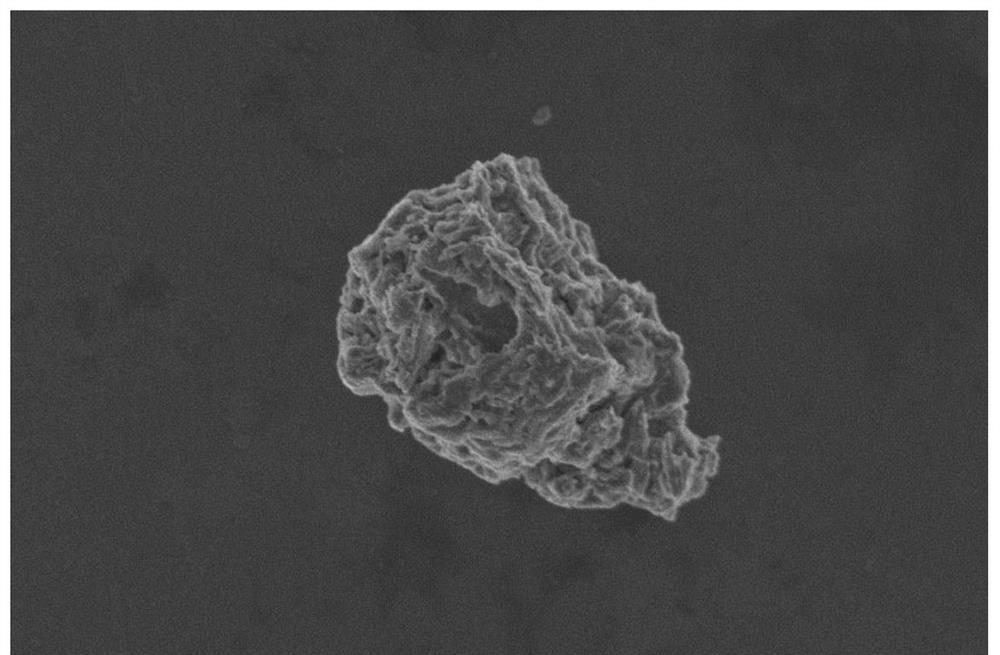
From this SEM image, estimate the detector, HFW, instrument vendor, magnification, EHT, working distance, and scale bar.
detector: TLD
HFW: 8.29 µm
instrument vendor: FEI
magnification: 50 K X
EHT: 2 kV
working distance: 4 mm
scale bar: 2000 nm